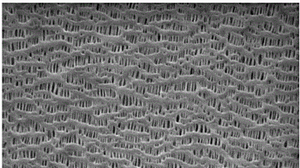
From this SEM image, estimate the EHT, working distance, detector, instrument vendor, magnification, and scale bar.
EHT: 10 kV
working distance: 6 mm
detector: SE1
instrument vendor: Zeiss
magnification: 20 K X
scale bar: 200 nm